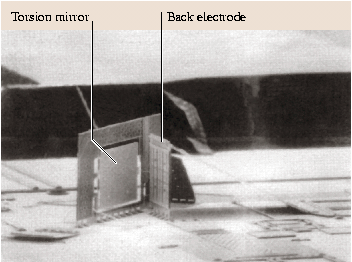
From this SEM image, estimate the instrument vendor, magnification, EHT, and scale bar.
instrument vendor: JEOL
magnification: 0.125 K X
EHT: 20 kV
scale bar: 100000 nm